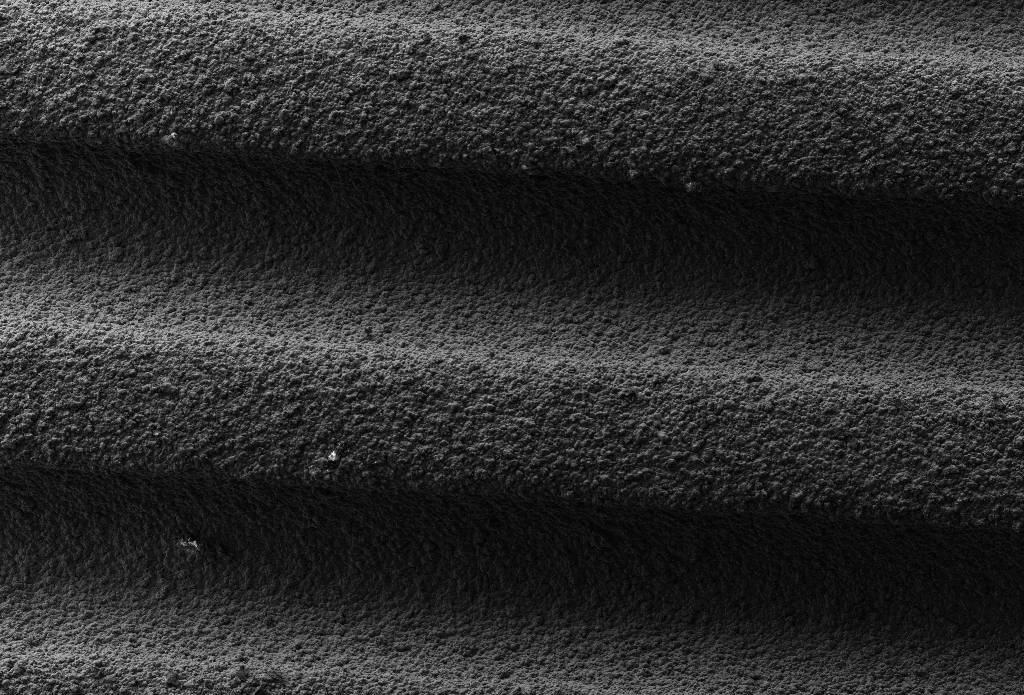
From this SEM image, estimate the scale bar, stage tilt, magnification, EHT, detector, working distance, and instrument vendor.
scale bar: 100000 nm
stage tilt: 0°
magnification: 0.124 K X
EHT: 5 kV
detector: SE2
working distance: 7.2 mm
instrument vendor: Zeiss